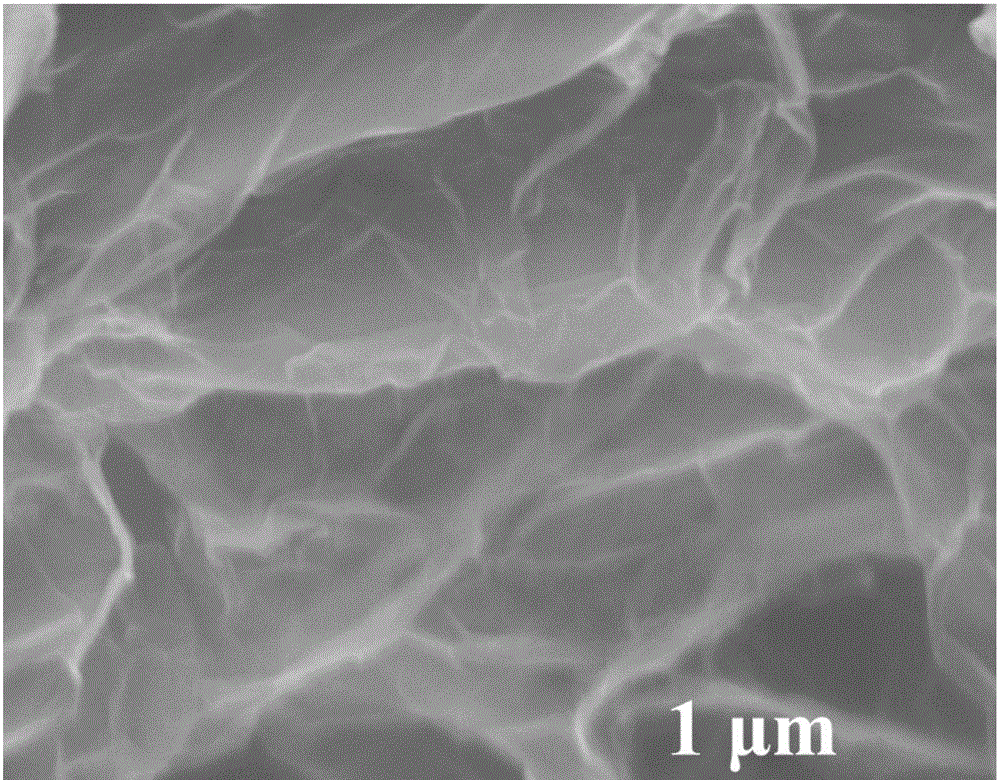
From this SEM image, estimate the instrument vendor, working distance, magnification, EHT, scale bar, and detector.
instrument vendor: Hitachi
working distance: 9.6 mm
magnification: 50 K X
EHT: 5 kV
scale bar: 1000 nm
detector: SE(M)